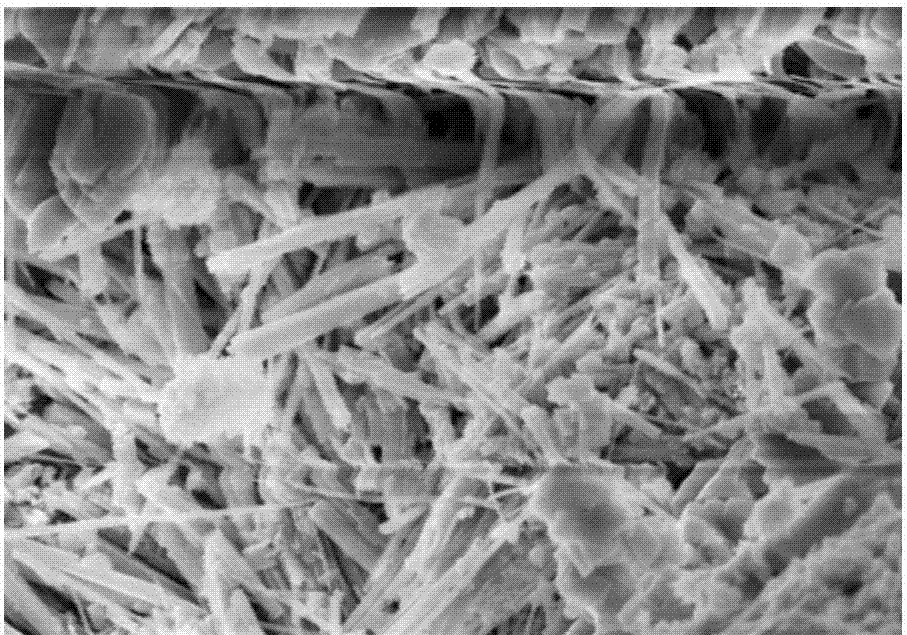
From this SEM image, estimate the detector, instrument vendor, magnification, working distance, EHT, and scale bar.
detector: SE(M,LA0)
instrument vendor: Hitachi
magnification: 25 K X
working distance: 8.3 mm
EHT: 5 kV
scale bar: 2000 nm